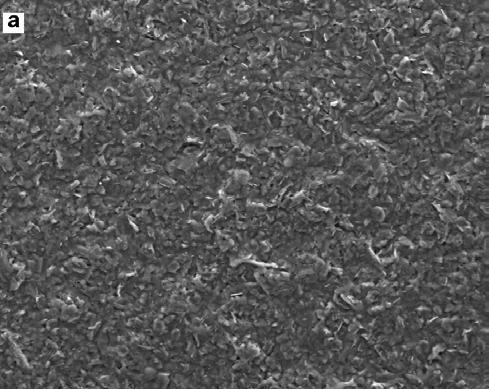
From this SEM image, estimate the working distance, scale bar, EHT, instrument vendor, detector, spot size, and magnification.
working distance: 9.3 mm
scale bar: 200000 nm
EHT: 20 kV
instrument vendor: FEI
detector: ETD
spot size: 4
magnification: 0.5 K X